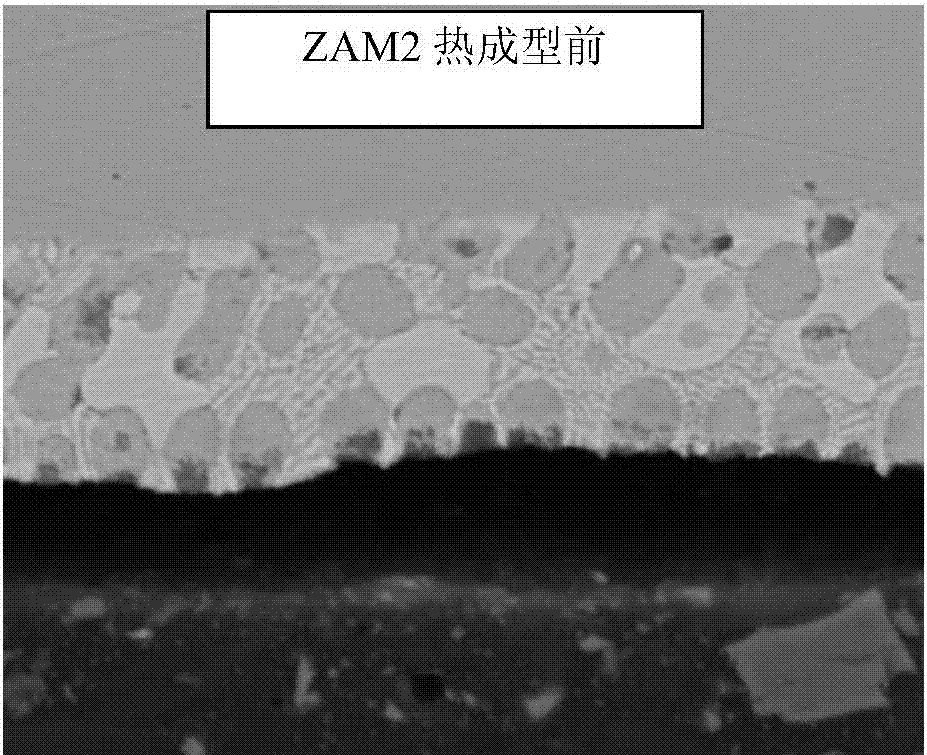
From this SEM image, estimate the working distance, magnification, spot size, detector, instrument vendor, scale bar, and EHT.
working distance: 8 mm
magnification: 2 K X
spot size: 4.5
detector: SSD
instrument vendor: FEI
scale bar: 20000 nm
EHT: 20 kV